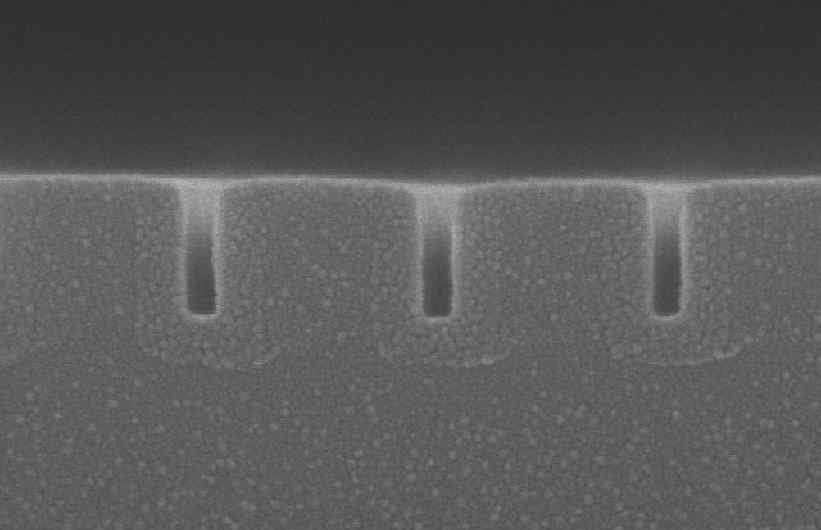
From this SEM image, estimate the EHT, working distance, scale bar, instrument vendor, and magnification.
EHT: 10 kV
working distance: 9.1 mm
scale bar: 400 nm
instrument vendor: Hitachi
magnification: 120 K X